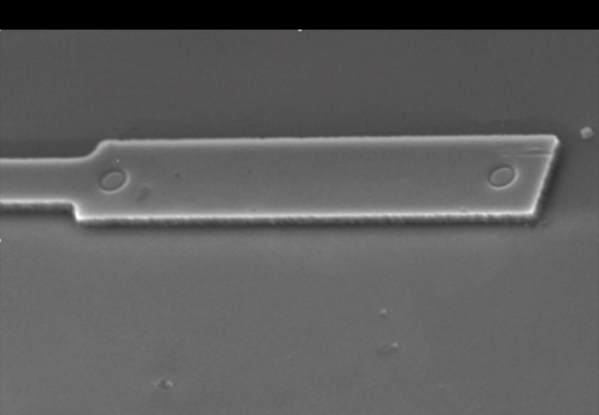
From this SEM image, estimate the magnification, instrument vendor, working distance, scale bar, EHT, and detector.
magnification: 5.5 K X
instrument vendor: JEOL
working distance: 10 mm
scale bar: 1000 nm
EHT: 15 kV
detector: LED